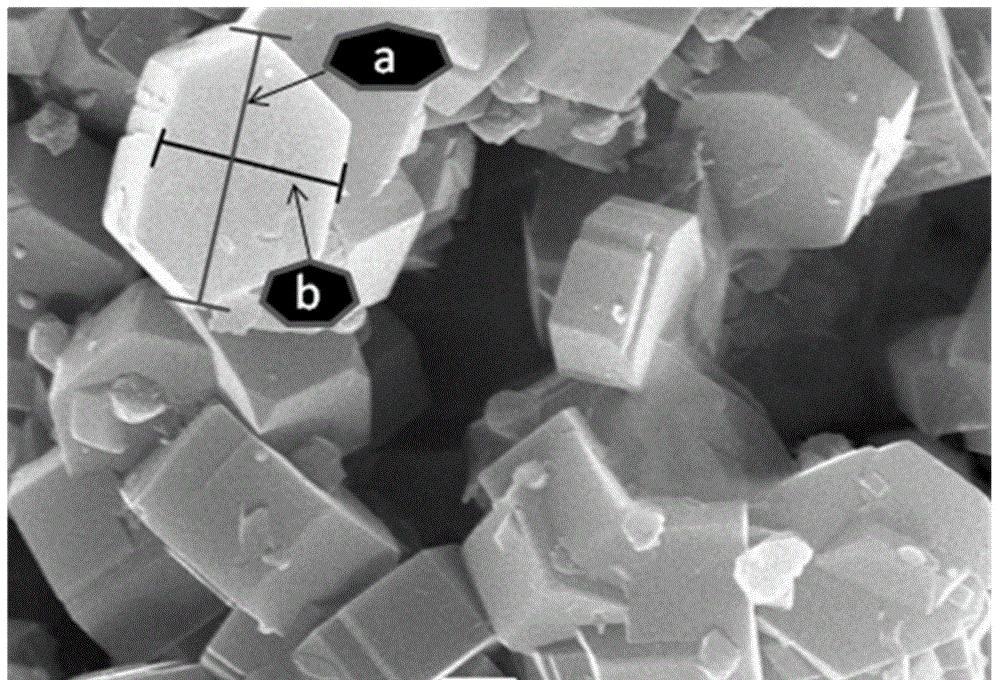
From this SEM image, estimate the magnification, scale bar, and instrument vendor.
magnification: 10 K X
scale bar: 1000 nm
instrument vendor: JEOL